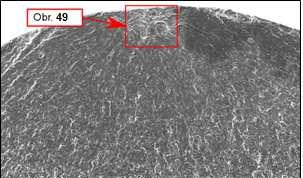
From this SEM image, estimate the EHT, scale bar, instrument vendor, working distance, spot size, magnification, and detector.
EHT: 20 kV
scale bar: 500000 nm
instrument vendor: FEI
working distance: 13 mm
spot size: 4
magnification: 0.05 K X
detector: SE